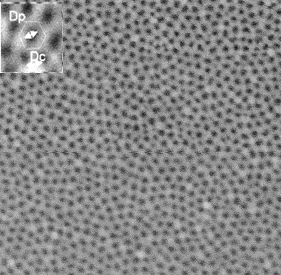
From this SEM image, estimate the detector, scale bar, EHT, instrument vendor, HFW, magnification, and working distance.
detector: SE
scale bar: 200 nm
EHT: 14 kV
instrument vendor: Tescan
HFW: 1.38 µm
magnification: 100 K X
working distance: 2.83 mm